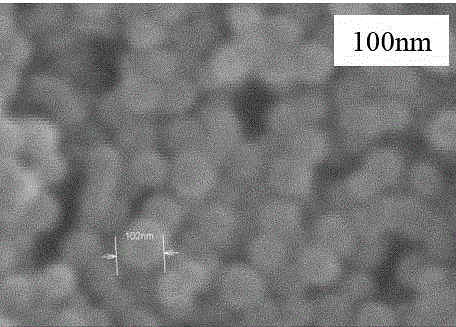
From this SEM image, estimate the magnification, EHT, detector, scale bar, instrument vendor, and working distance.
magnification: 130 K X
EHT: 30 kV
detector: SE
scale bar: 400 nm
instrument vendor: Hitachi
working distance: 8.7 mm